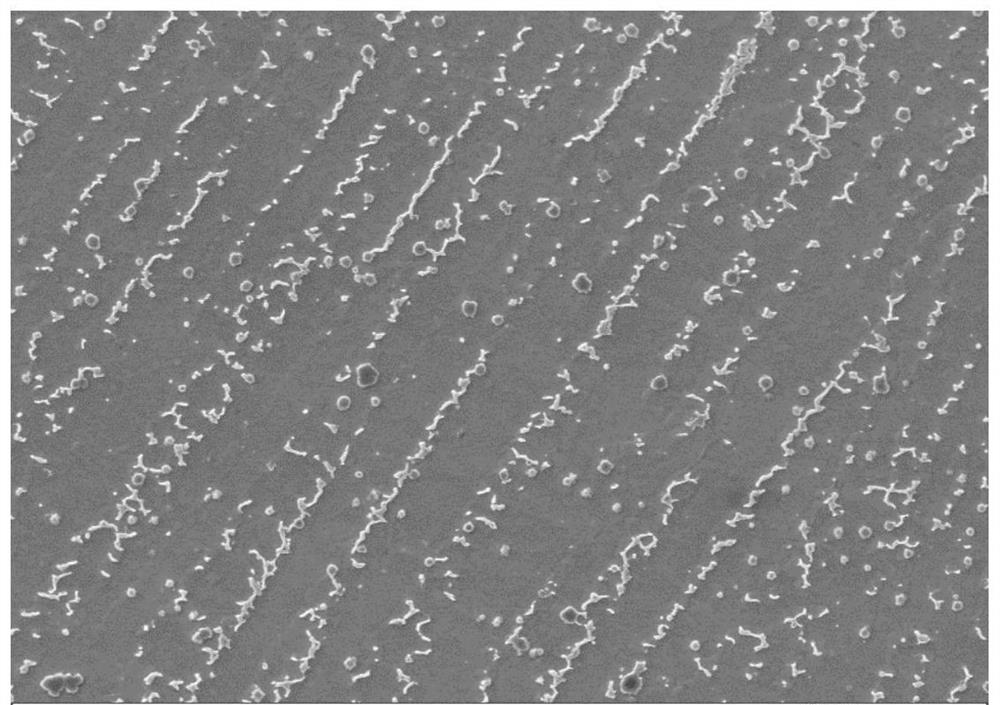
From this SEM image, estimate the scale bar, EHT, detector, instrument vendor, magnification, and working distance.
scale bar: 10000 nm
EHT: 20 kV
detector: SE1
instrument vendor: Zeiss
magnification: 2 K X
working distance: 9 mm